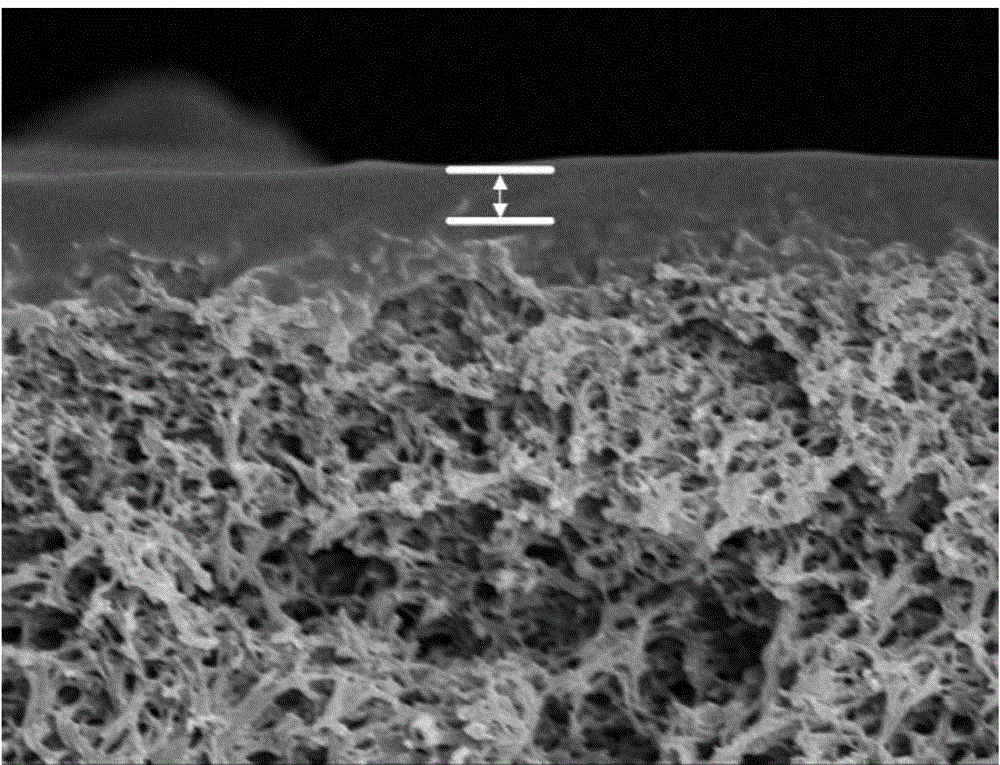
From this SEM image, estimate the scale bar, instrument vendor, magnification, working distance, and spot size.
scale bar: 3000 nm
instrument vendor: FEI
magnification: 20 K X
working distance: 5.2 mm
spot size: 3